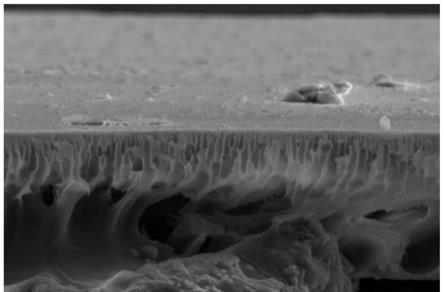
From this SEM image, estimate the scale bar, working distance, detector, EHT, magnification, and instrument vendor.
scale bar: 50000 nm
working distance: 5.4 mm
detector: ETD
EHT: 15 kV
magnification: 3 K X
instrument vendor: FEI